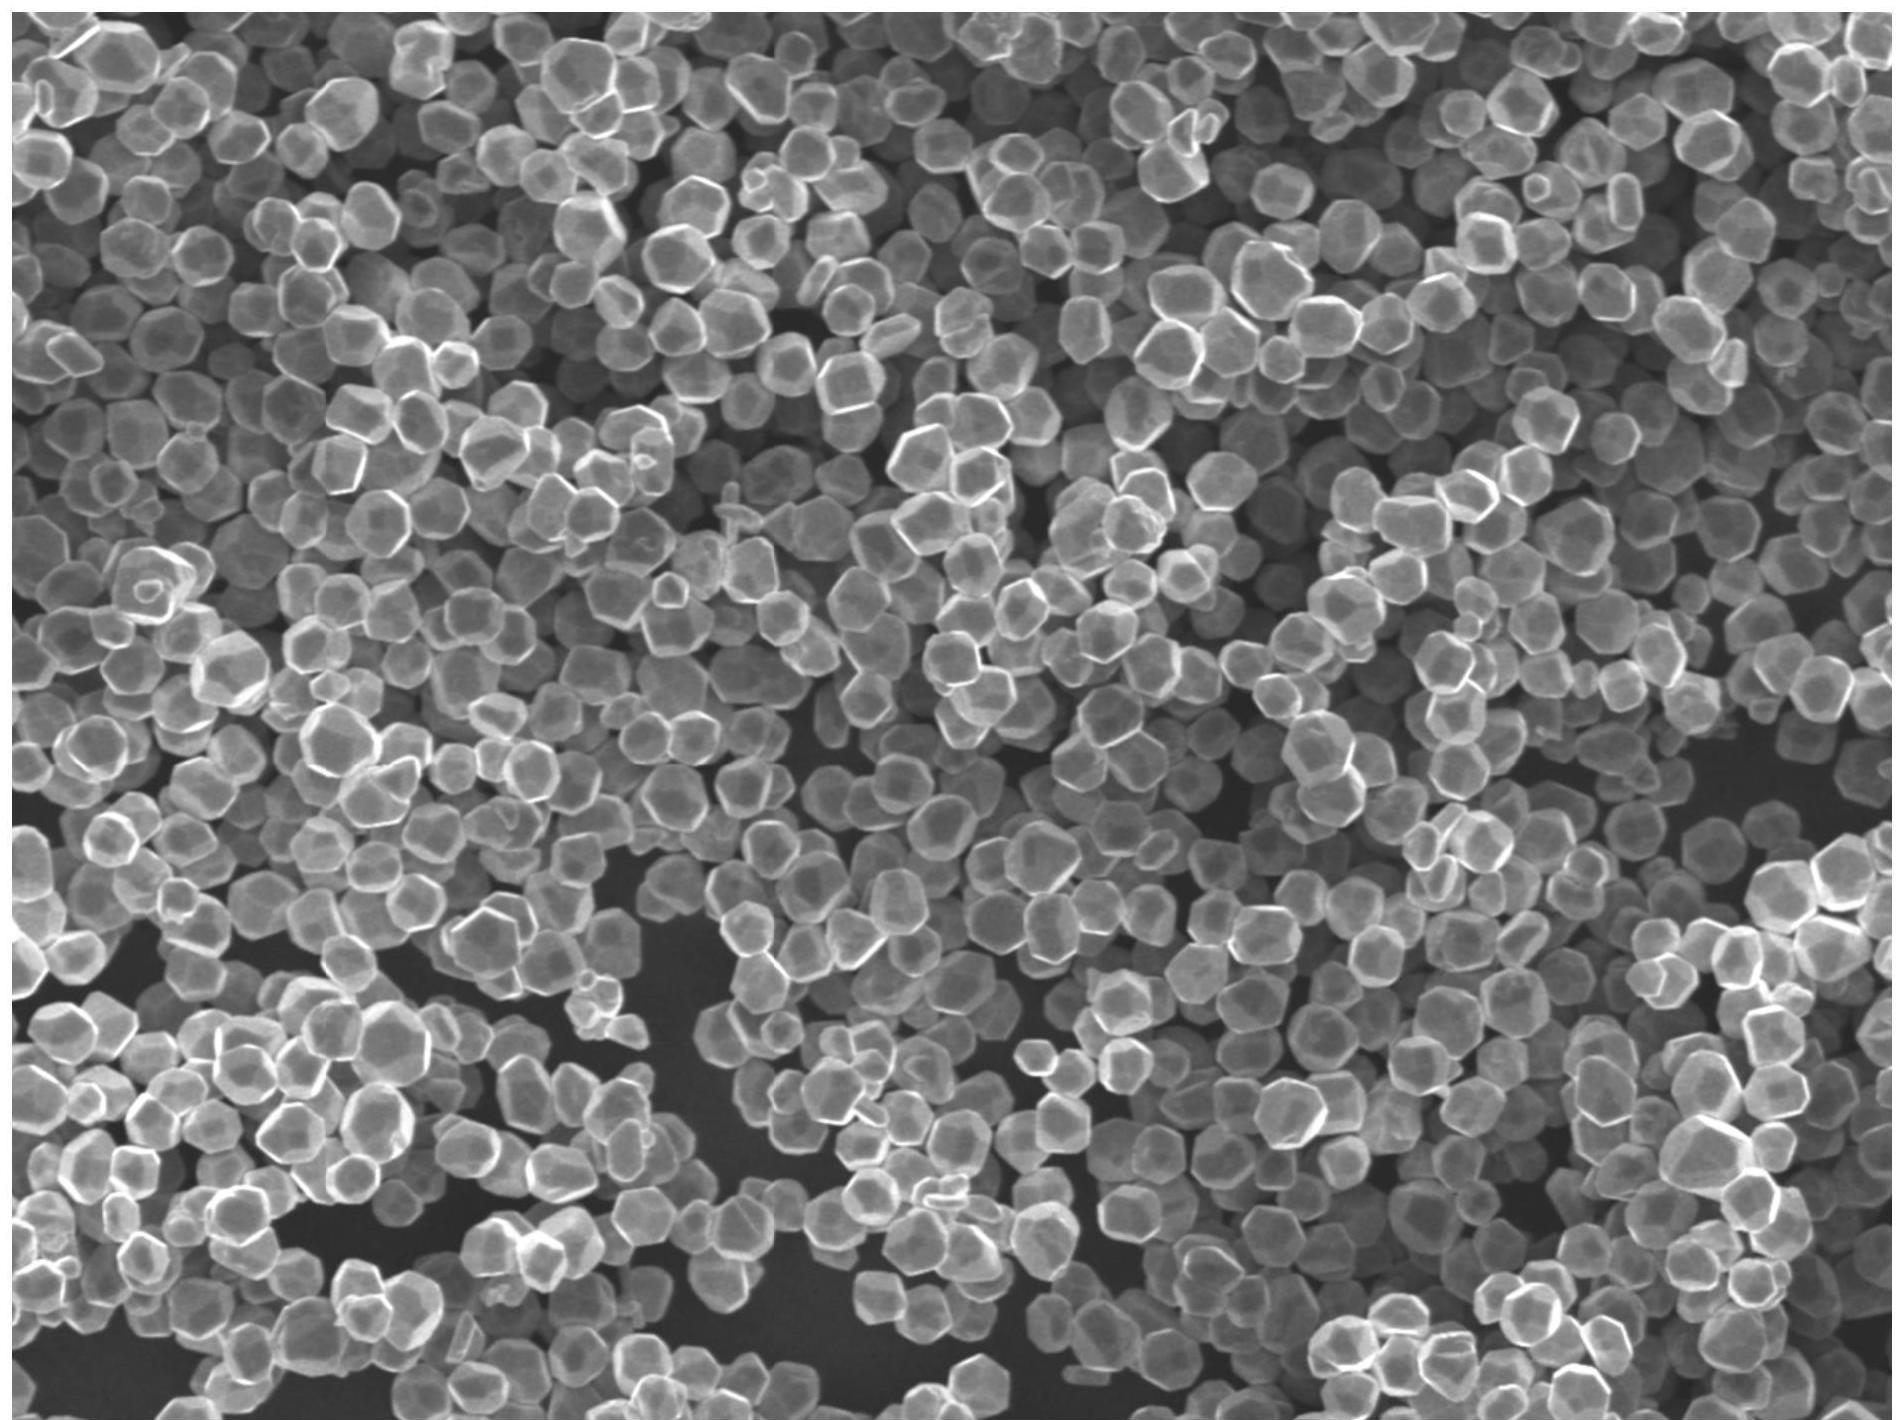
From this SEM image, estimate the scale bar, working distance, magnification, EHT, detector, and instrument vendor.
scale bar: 10000 nm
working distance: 10 mm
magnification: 1 K X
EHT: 15 kV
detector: SEI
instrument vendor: JEOL